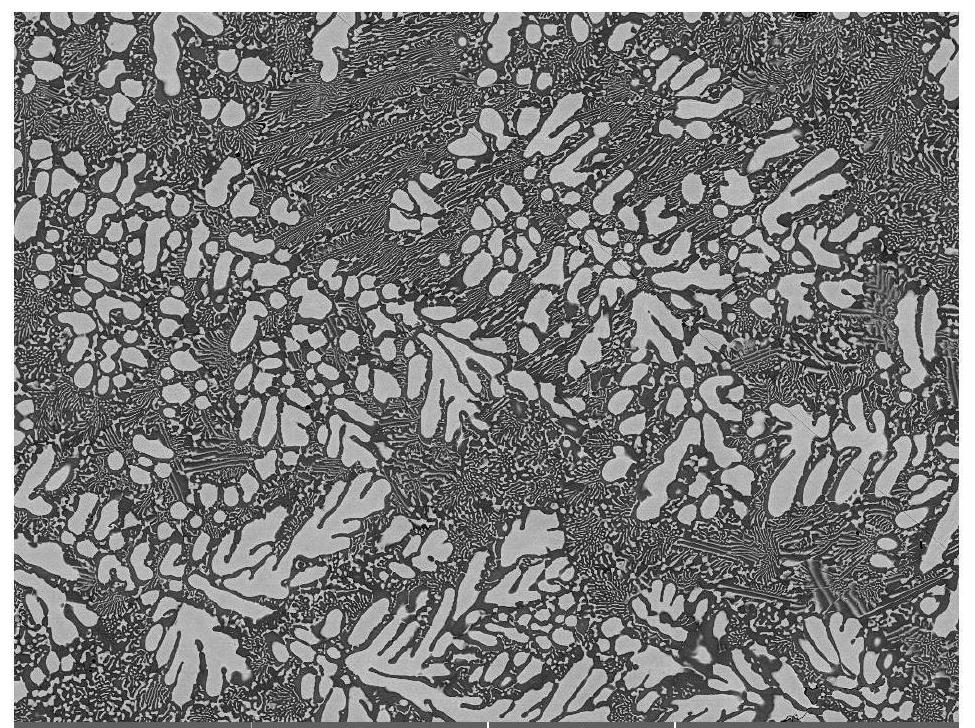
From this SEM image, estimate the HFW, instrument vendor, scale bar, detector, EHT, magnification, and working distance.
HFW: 252 µm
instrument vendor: Tescan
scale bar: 50000 nm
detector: LE-BSE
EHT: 20 kV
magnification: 1.1 K X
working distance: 15.01 mm